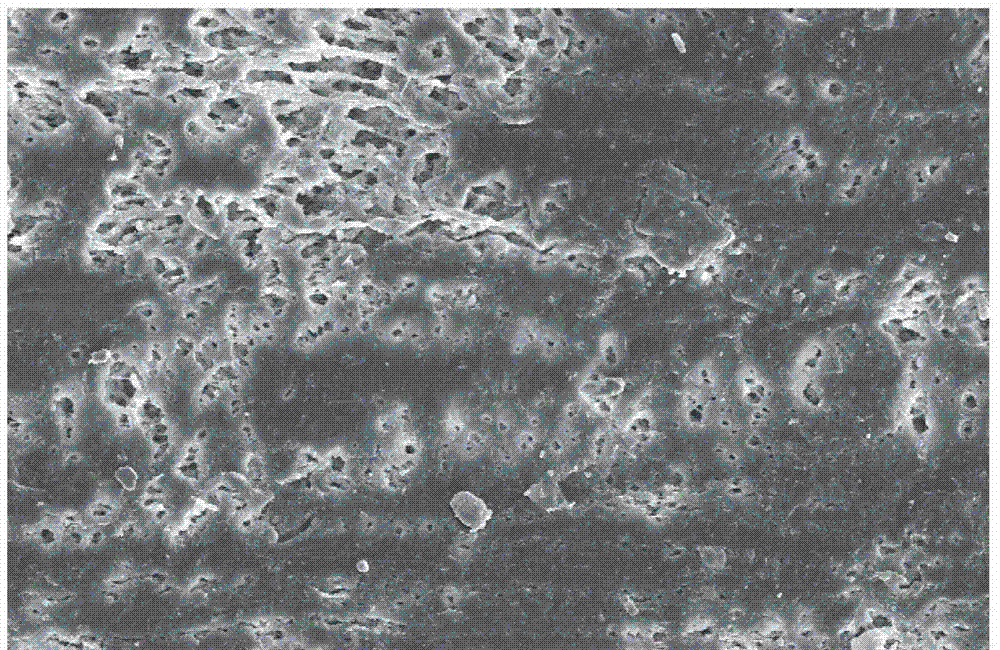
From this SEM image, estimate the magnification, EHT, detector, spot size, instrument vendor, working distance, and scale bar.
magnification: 0.5 K X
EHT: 10 kV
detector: SE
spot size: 3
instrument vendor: FEI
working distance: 10.6 mm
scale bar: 50000 nm